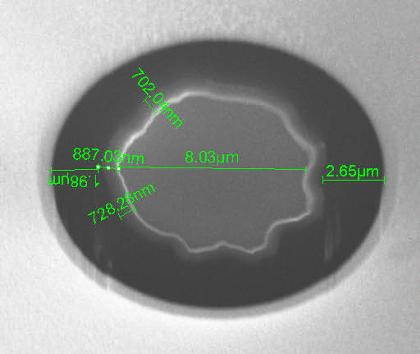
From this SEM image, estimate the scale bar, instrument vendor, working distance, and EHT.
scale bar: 5000 nm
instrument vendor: FEI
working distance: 15 mm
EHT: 20 kV